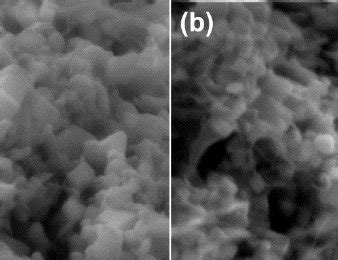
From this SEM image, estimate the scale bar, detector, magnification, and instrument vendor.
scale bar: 200 nm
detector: SE2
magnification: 20 K X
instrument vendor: Zeiss